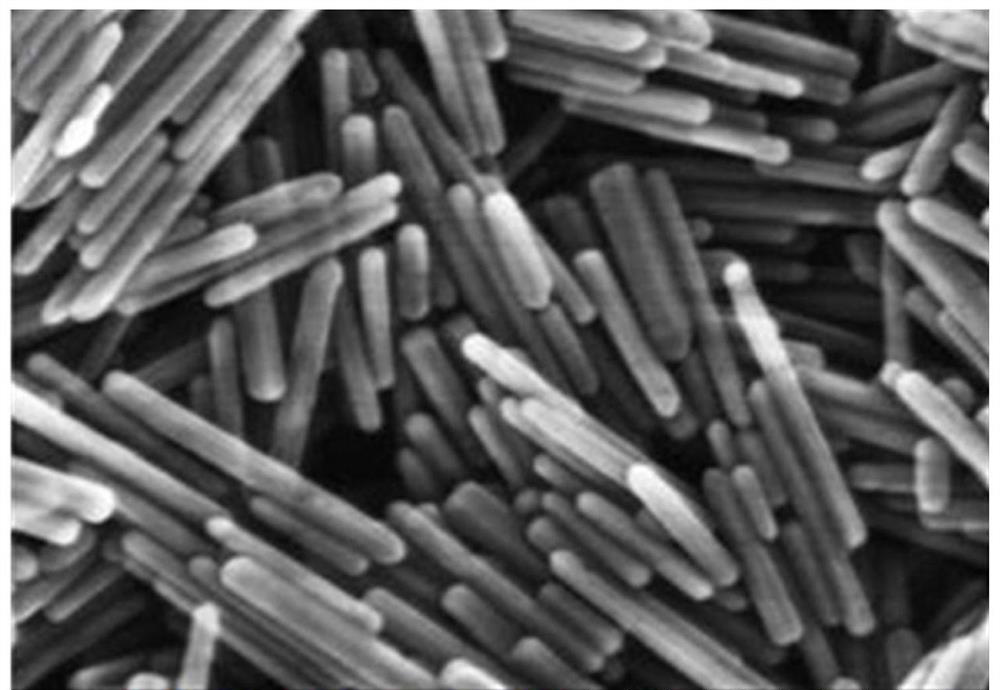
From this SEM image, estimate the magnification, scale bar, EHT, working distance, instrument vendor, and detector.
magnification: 220 K X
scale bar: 200 nm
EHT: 20 kV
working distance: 9 mm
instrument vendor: Hitachi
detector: SE(U)